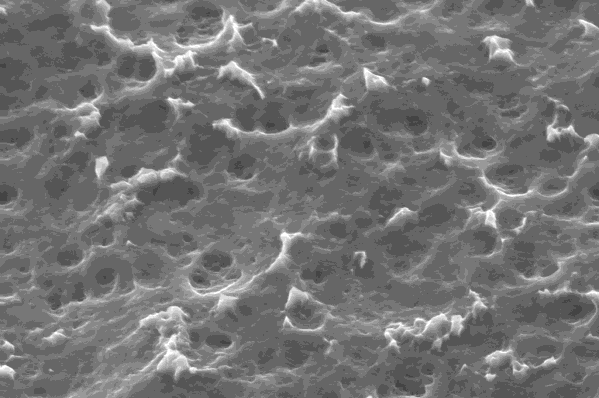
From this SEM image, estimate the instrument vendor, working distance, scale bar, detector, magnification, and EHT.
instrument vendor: Zeiss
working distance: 11 mm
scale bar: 2000 nm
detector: SE1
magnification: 5 K X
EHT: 20 kV